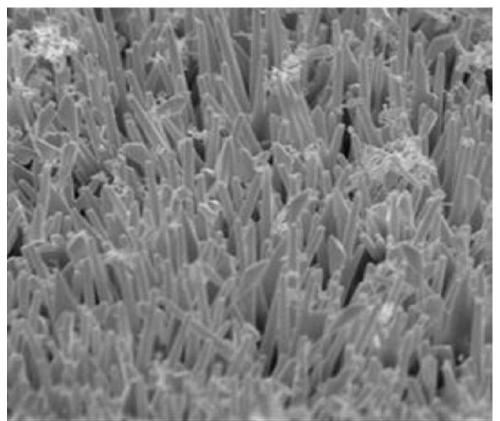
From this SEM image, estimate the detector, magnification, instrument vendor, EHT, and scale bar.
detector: SEI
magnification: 3.7 K X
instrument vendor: JEOL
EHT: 20 kV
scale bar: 1000 nm